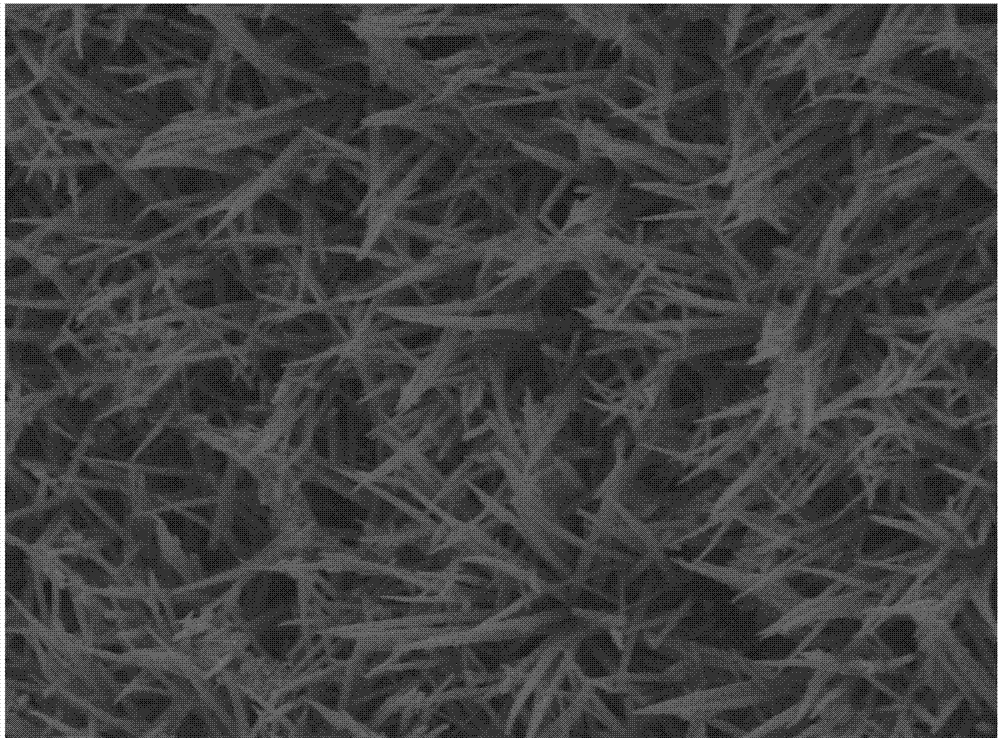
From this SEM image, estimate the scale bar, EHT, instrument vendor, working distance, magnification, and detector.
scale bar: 1000 nm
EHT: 5 kV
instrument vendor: JEOL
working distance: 10 mm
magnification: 10 K X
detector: LED